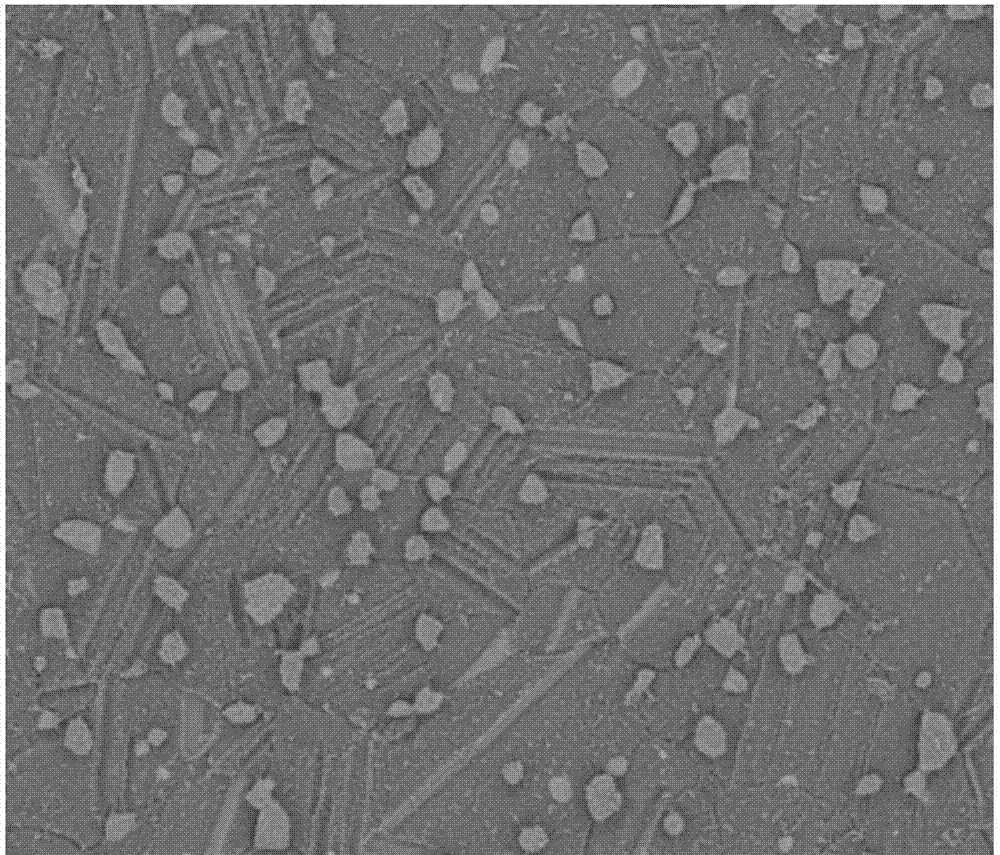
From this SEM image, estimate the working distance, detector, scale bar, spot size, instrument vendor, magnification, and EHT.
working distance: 6.4 mm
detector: vCD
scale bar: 10000 nm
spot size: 3.5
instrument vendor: FEI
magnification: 10 K X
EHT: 3 kV